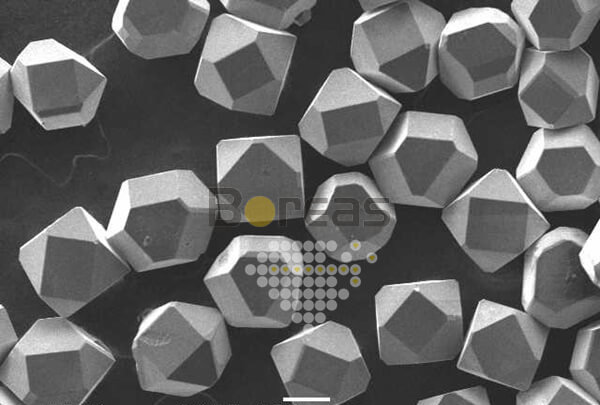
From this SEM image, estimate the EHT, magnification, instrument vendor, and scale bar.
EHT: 20 kV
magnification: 0.1 K X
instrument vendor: JEOL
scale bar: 100000 nm